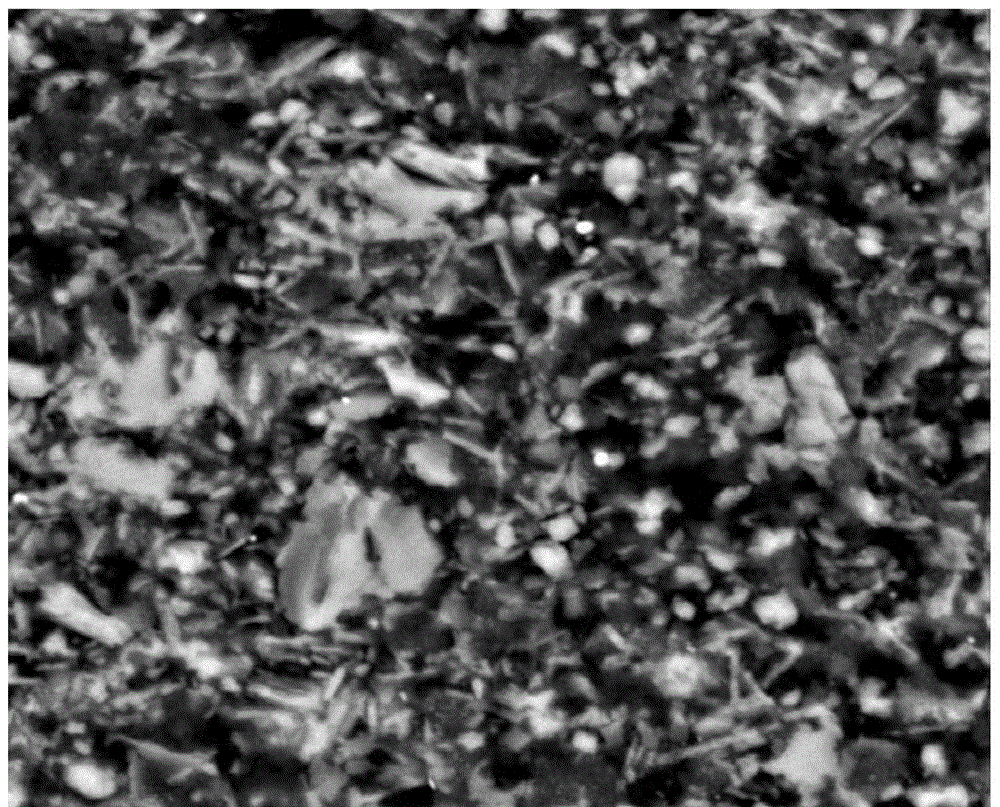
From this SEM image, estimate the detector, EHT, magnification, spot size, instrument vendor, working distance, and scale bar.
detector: SSD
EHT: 20 kV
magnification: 2.5 K X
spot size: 4.5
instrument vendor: FEI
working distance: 10.3 mm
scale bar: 20000 nm